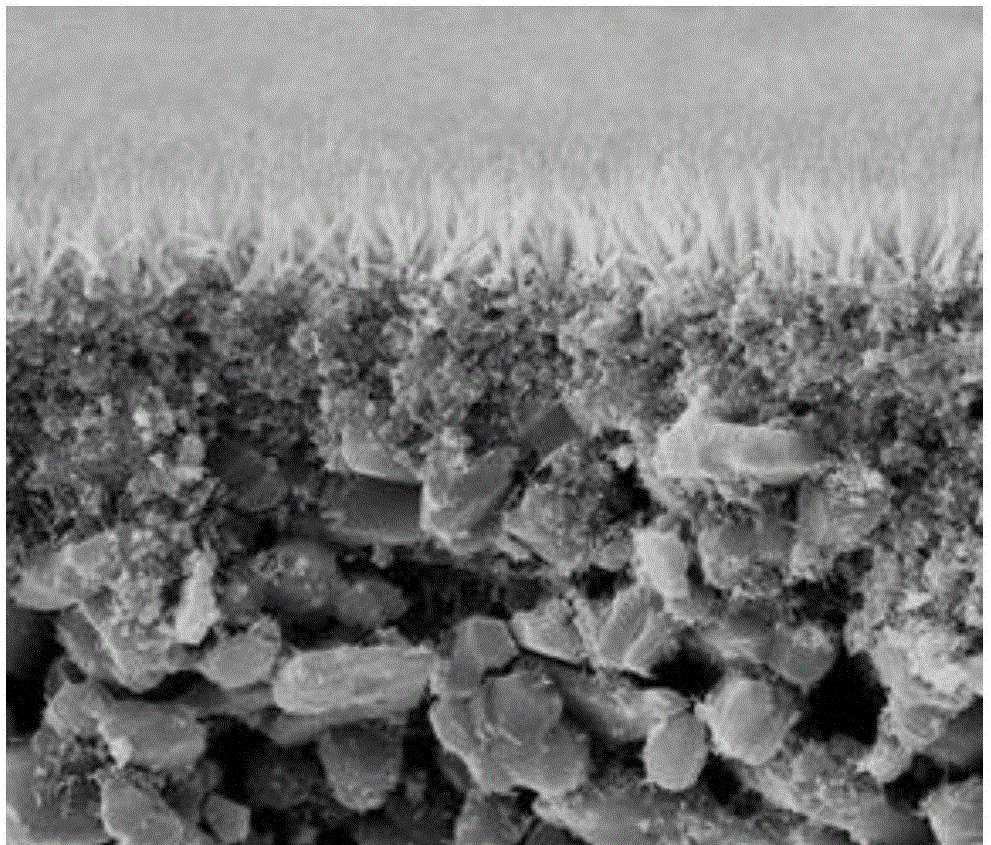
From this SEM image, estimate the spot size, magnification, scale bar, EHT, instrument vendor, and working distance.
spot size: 2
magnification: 10 K X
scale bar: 10000 nm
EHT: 20 kV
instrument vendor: FEI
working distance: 9.7 mm